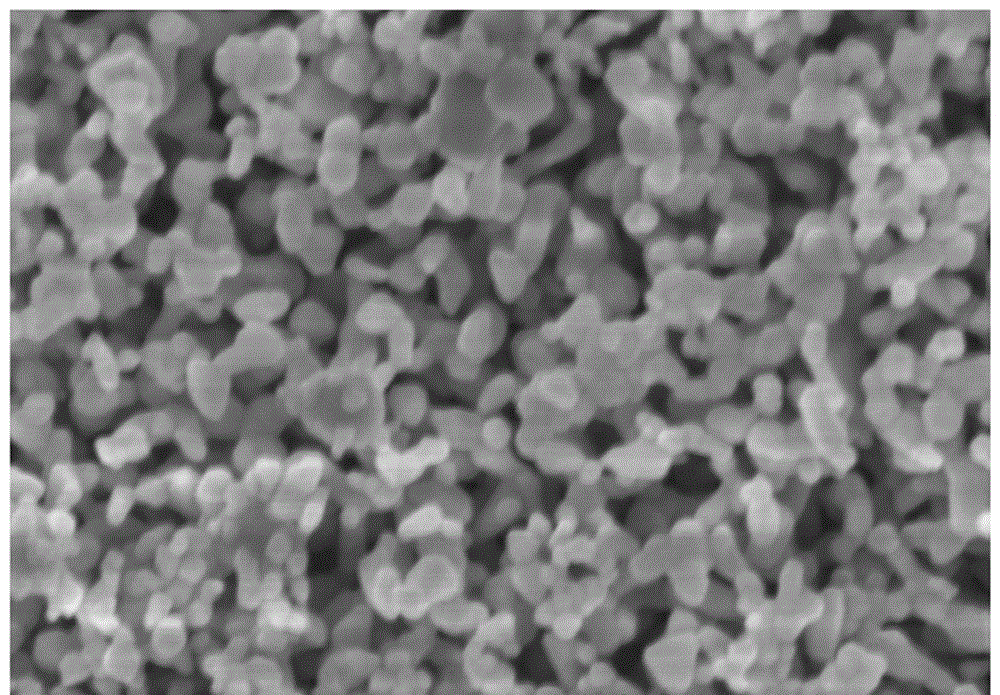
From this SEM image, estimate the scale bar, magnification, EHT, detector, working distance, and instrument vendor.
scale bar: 500 nm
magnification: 100 K X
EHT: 3 kV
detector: SE(M)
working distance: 4.4 mm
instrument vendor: Hitachi